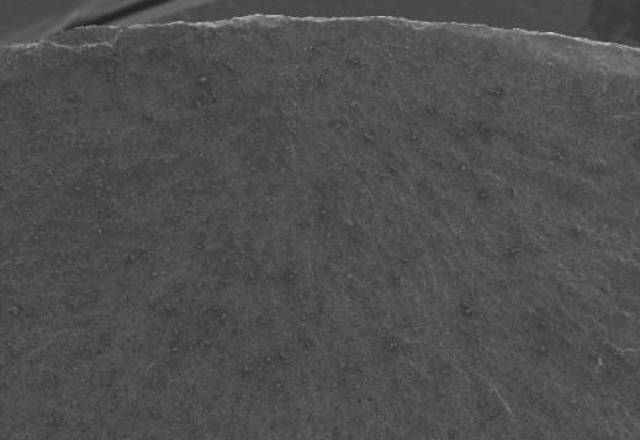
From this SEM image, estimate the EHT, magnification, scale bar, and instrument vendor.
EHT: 20 kV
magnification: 0.02 K X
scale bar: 1000000 nm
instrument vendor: JEOL